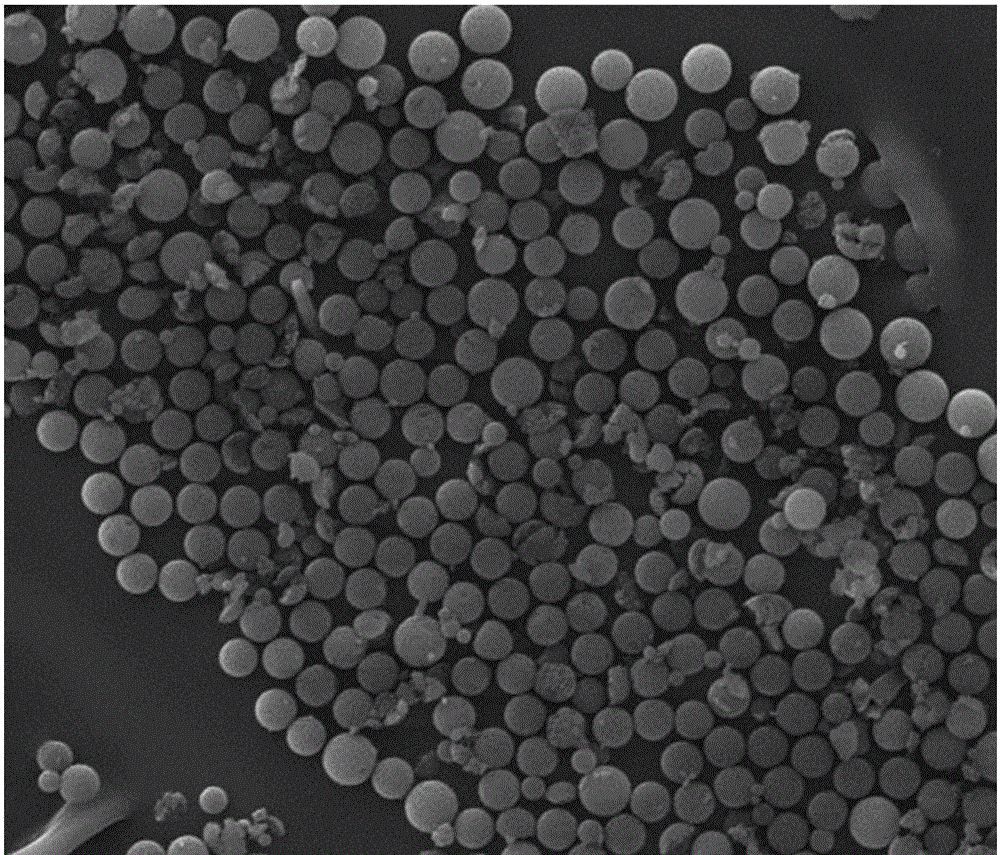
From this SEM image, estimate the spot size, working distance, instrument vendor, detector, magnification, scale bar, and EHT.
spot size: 4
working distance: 24.1 mm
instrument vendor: FEI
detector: ETD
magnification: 0.6 K X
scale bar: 50000 nm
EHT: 30 kV